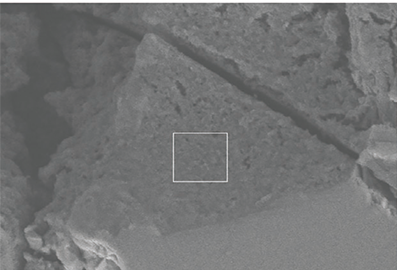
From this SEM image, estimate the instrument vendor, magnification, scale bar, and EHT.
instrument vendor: Hitachi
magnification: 11 K X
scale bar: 2730 nm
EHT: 4 kV